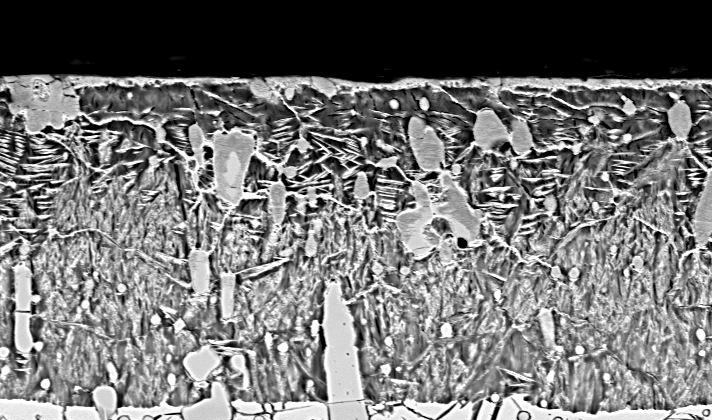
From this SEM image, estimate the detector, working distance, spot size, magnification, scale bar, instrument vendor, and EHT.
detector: BSE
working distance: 10.4 mm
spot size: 5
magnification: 1 K X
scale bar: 20000 nm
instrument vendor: FEI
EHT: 20 kV